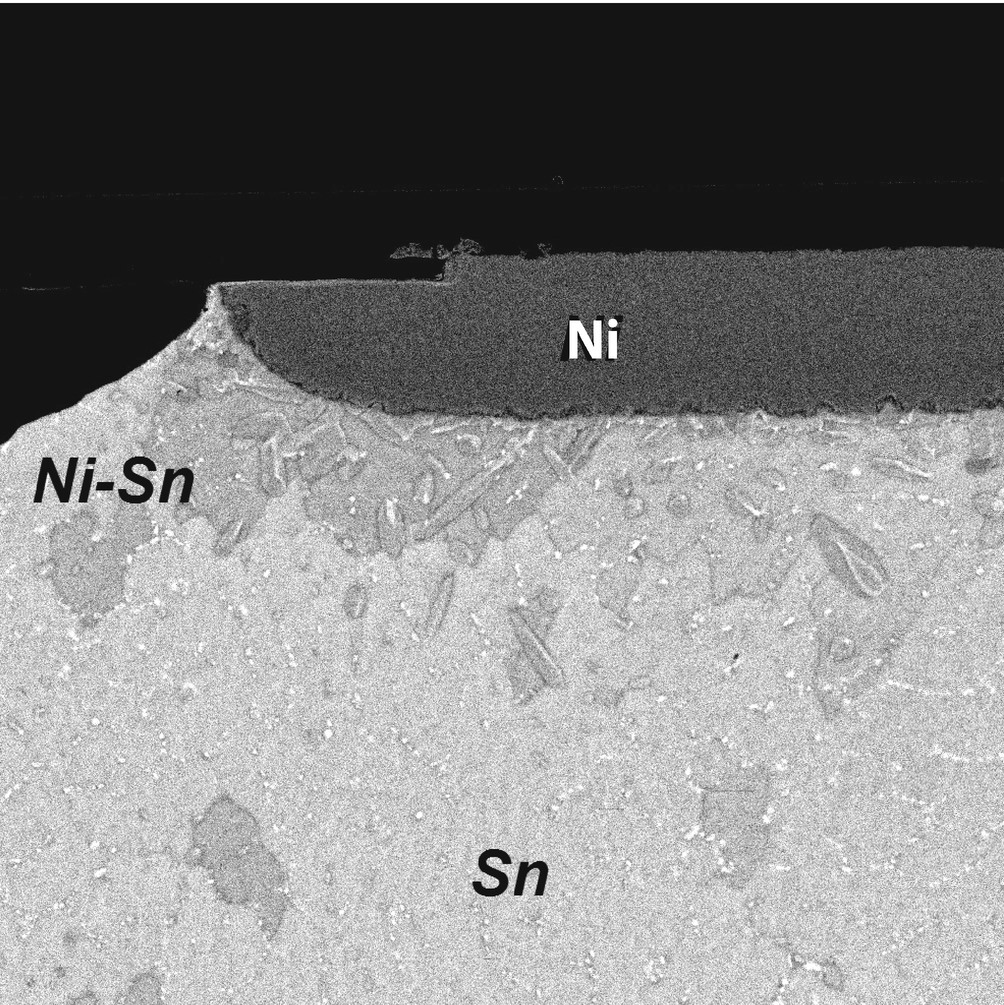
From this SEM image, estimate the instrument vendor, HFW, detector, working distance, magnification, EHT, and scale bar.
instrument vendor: Zeiss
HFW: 30 µm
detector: MCP RBI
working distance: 10.4 mm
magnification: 4.23333 K X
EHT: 32.9529 kV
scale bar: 2000 nm